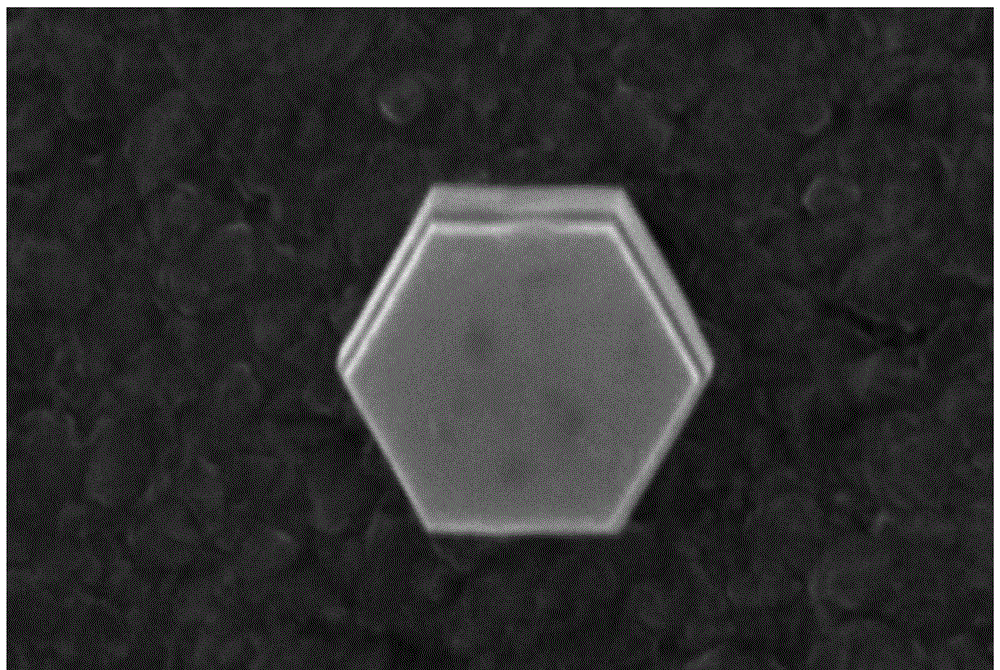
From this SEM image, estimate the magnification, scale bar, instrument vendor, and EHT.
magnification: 40 K X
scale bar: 500 nm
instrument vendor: JEOL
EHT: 30 kV